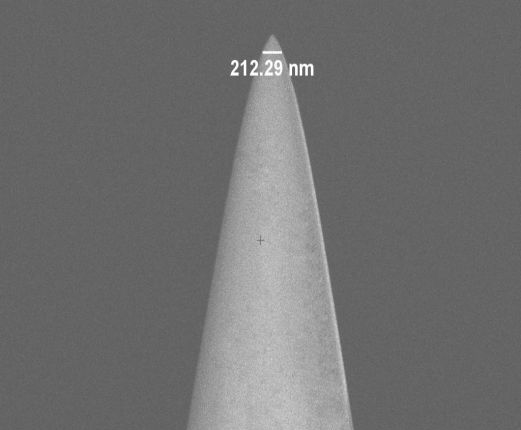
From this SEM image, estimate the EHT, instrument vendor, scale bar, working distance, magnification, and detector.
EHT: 2 kV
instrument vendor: Zeiss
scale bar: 2000 nm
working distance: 4.5 mm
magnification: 20 K X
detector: InLens